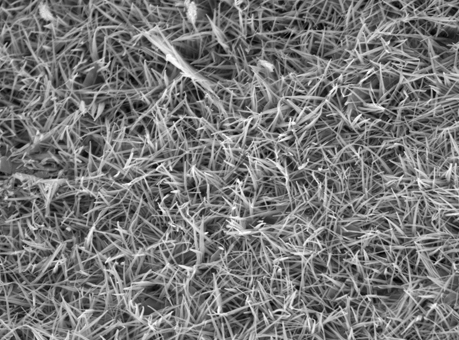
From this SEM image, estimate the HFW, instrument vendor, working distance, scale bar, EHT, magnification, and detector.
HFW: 12.8 µm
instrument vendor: JEOL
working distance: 10.9 mm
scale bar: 1000 nm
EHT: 10 kV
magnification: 10 K X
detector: SED